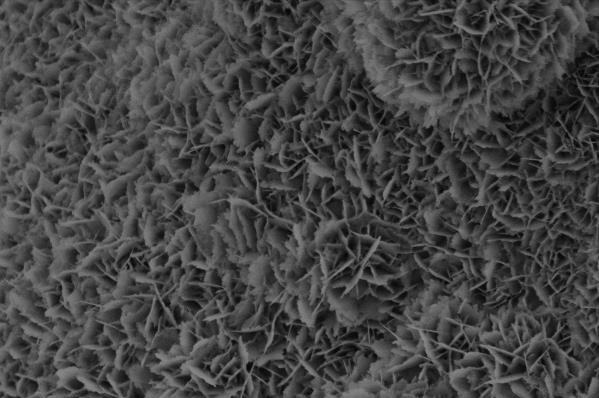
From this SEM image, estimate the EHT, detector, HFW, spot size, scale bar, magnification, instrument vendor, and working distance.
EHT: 2 kV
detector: ETD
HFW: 19 µm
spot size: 2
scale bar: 5000 nm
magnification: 21.806 K X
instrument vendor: FEI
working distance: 10.4 mm